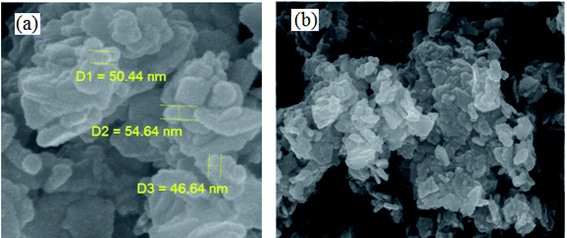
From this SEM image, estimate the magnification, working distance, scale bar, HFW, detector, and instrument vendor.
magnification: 200 K X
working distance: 5.05 mm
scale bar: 200 nm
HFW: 1.04 µm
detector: InBeam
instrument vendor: Tescan